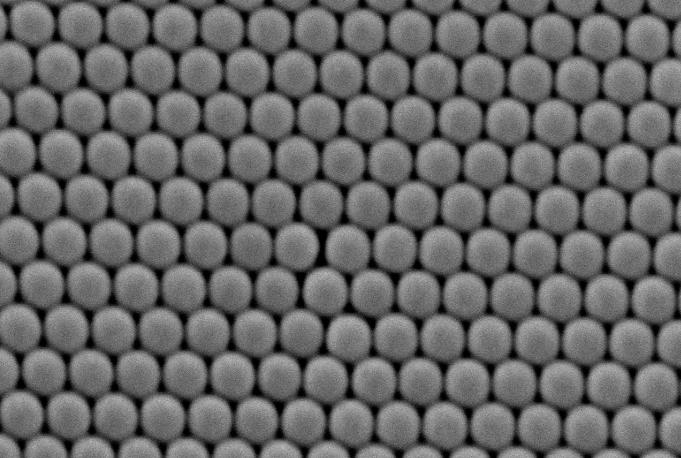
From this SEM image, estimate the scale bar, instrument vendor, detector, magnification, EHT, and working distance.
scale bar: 1000 nm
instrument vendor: JEOL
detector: SE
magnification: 40 K X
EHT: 15 kV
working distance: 9 mm